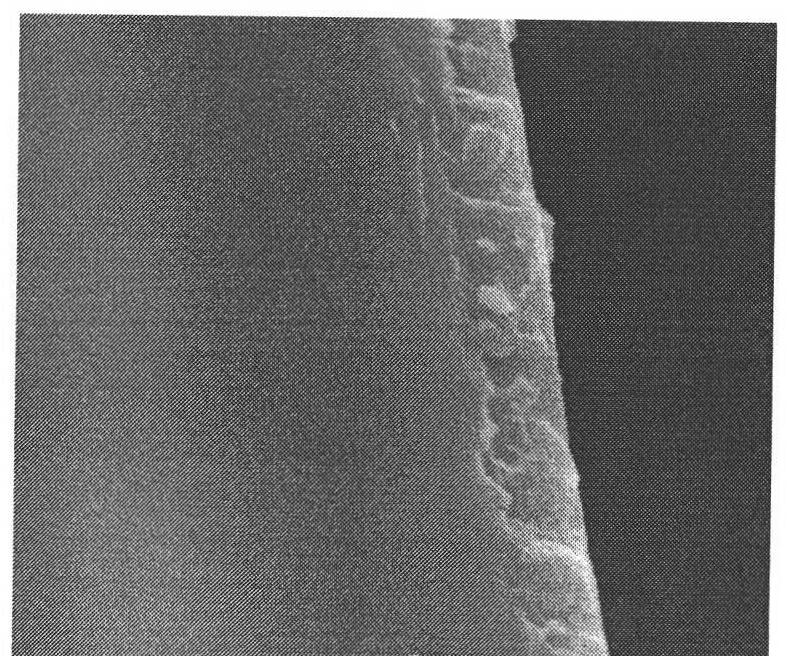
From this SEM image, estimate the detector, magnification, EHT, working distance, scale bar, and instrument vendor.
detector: ETD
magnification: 40 K X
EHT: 20 kV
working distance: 11.7 mm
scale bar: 2000 nm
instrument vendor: FEI